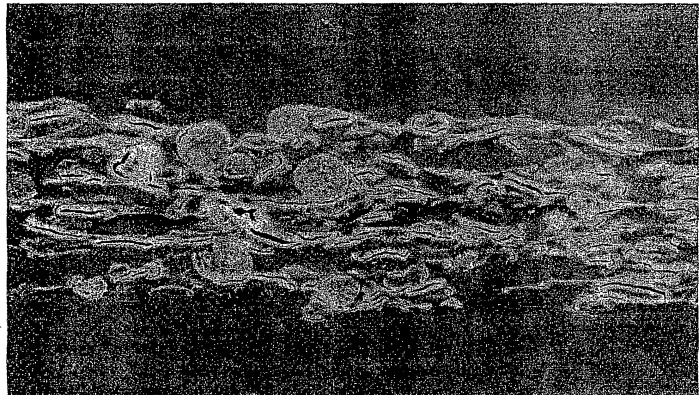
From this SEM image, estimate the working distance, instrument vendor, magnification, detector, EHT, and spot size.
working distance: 17 mm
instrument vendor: FEI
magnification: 0.2 K X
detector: SE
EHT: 20 kV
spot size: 4